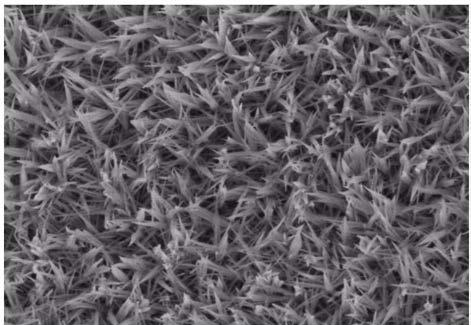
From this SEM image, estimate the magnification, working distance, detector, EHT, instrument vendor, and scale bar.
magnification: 20 K X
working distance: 11 mm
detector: SE(UL)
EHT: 3 kV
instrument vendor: Hitachi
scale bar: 2000 nm